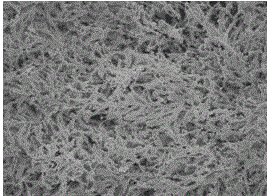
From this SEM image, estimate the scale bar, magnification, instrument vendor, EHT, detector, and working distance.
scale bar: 1000 nm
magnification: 20 K X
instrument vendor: JEOL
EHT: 10 kV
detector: SEI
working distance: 7.5 mm